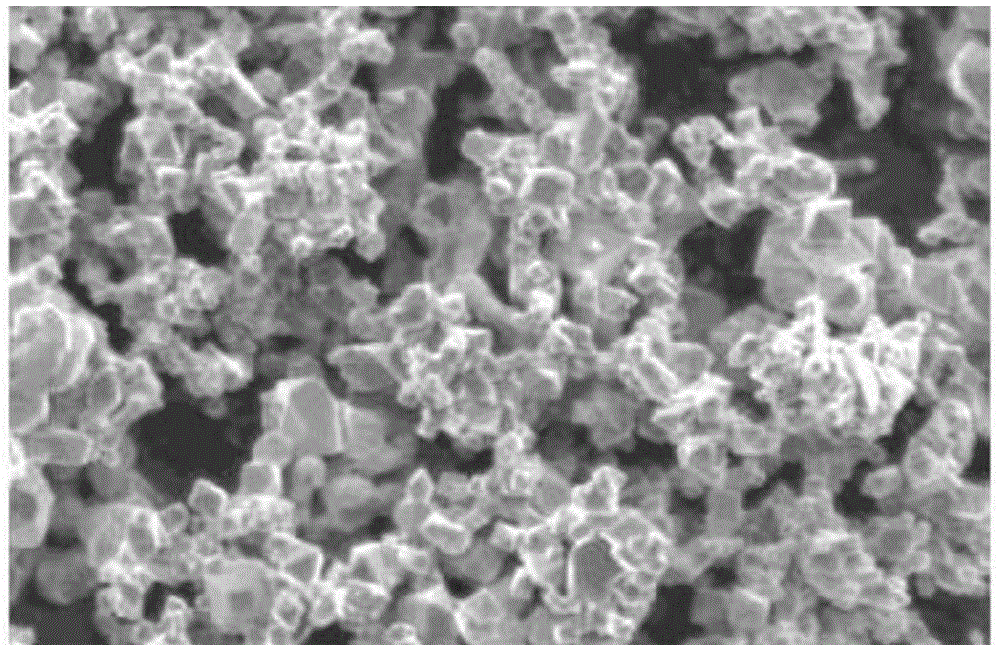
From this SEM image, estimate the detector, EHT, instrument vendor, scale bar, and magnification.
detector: SEI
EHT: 5 kV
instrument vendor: JEOL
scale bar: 5000 nm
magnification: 5 K X